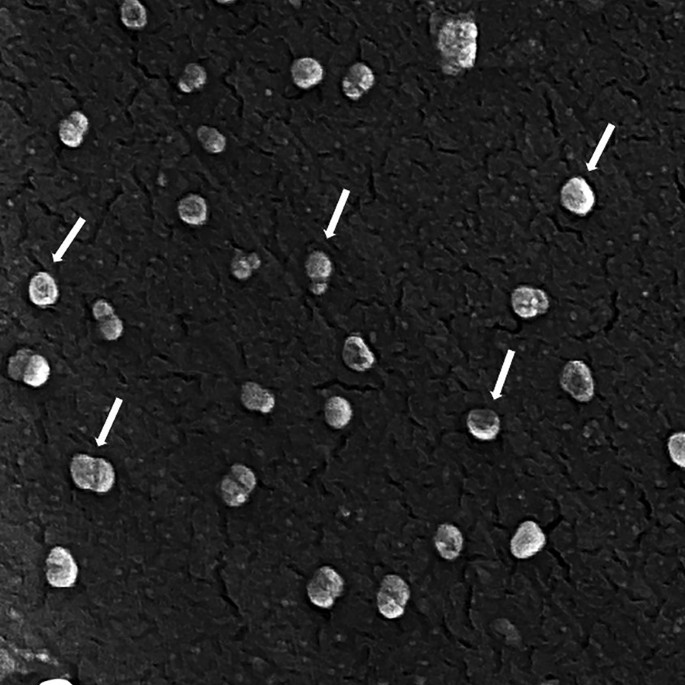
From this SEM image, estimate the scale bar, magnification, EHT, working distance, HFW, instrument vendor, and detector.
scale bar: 500 nm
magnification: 70 K X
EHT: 10 kV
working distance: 4.73 mm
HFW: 1.98 µm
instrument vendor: Tescan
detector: InBeam